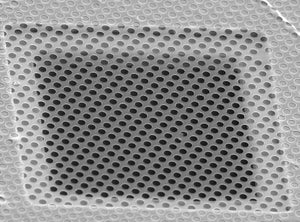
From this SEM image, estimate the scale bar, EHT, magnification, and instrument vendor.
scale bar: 20000 nm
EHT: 25 kV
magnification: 1.5 K X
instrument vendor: other